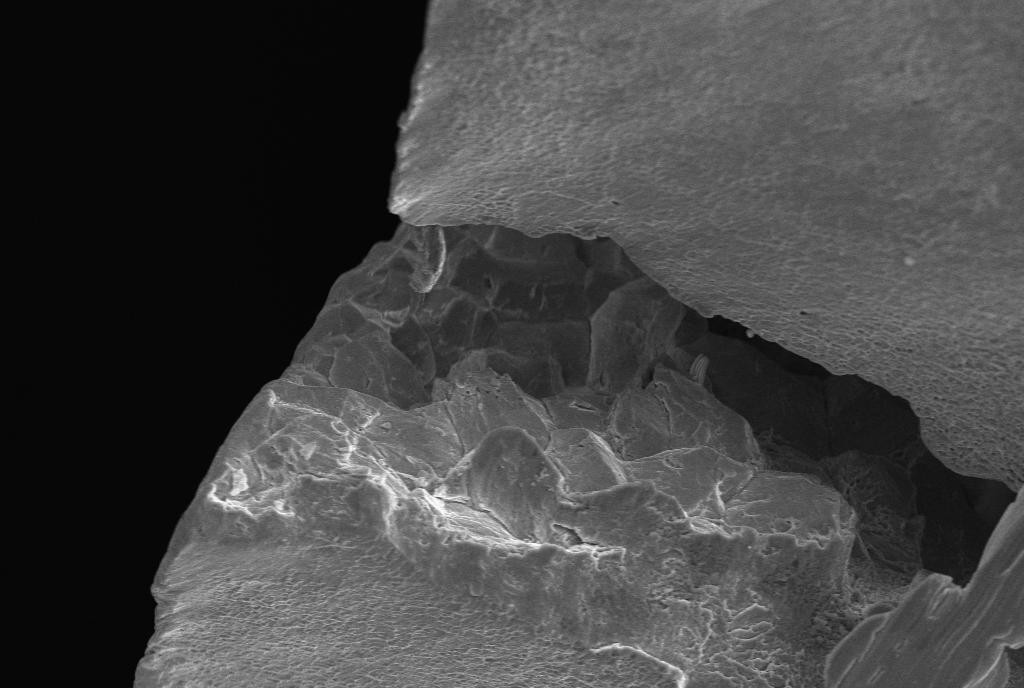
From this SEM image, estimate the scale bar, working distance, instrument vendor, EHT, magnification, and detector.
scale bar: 20000 nm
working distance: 18.5 mm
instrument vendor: Zeiss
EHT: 20 kV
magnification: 0.5 K X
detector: SE1